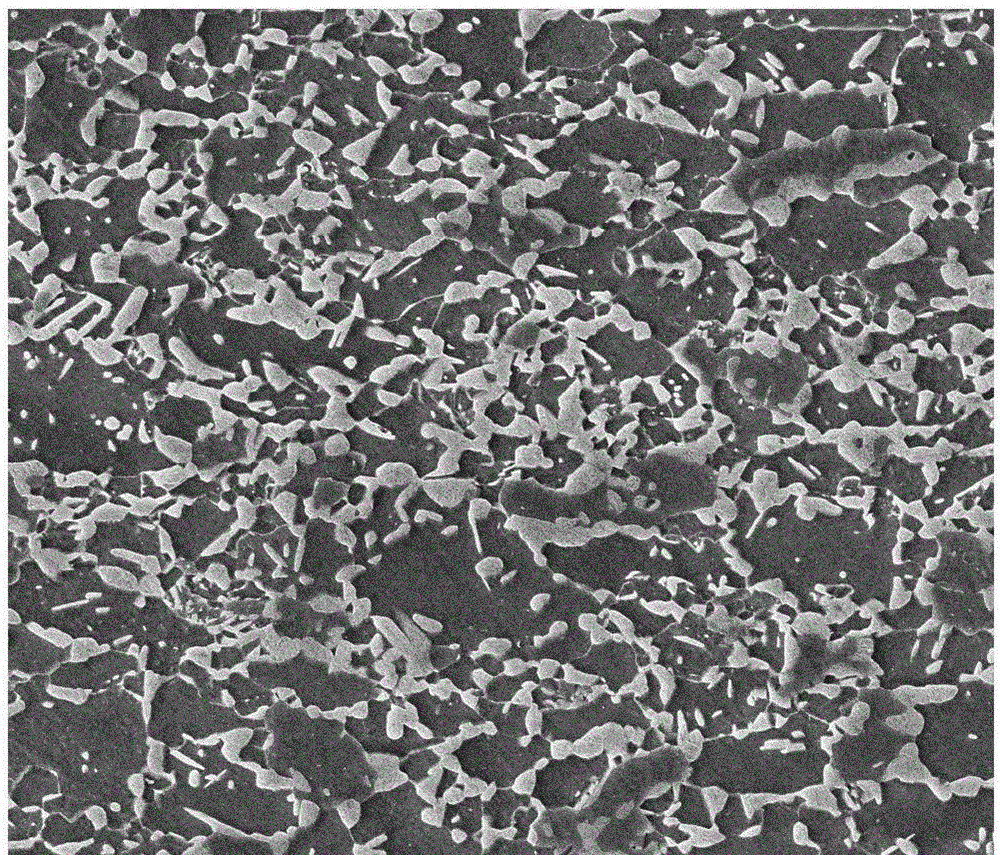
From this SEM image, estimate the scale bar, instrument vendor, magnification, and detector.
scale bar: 40000 nm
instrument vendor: FEI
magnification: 5 K X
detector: ETD